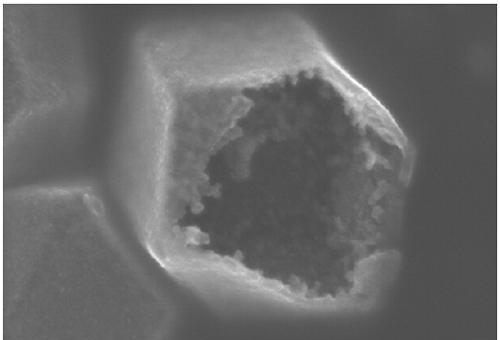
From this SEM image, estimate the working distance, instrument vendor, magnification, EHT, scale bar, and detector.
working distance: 9 mm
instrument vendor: Hitachi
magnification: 70 K X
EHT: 15 kV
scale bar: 500 nm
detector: SE(U)